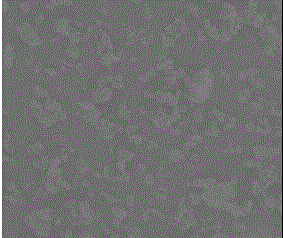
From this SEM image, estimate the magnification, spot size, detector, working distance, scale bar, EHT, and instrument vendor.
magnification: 0.209 K X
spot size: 5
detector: LFD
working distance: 11.7 mm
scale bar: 200000 nm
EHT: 10 kV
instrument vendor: FEI